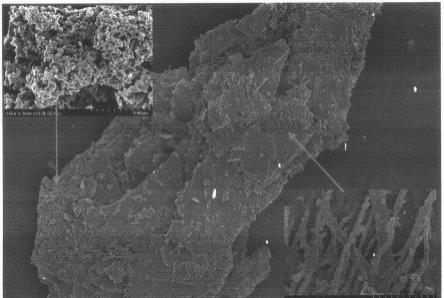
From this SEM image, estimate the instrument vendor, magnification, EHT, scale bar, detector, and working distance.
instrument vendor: Hitachi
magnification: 2 K X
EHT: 3 kV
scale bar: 20000 nm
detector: SE(UL)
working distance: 8.3 mm